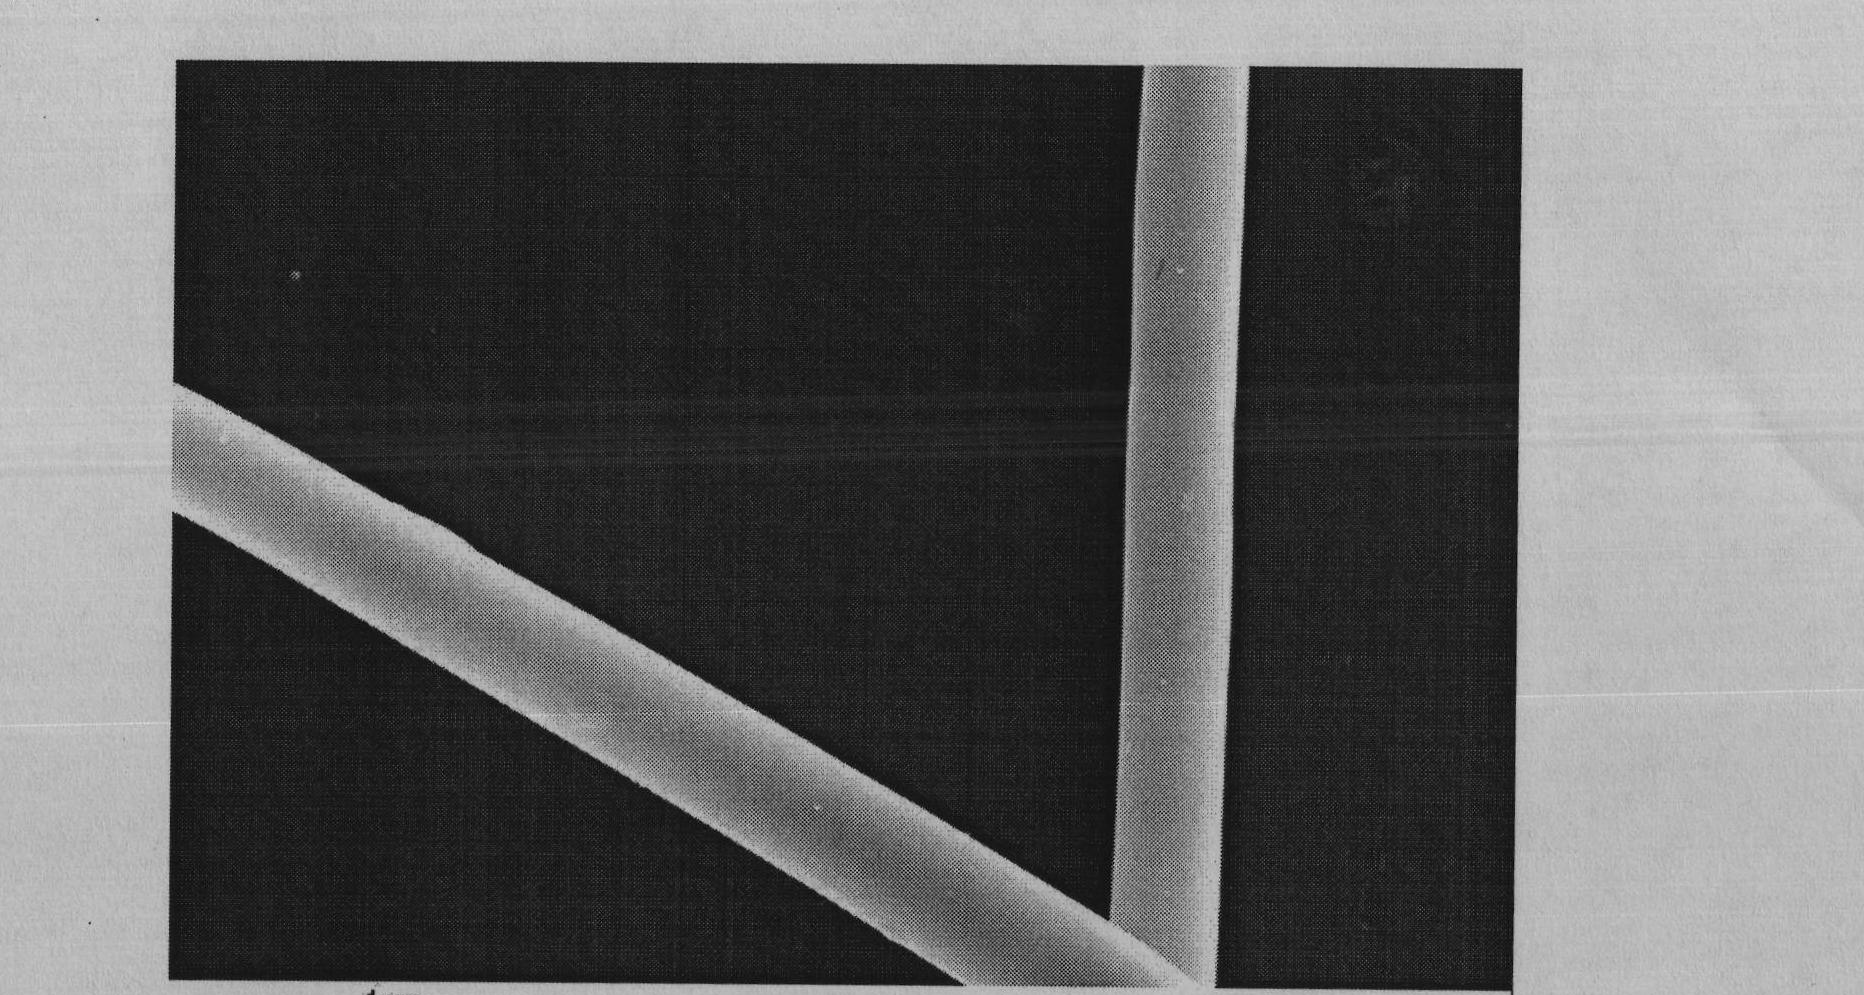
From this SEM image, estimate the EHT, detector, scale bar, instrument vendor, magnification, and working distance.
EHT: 20 kV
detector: InLens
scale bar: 1000 nm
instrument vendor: Zeiss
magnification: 3 K X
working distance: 3.6 mm